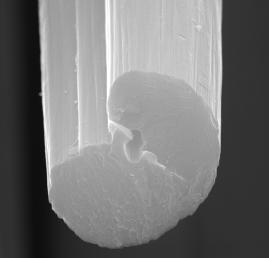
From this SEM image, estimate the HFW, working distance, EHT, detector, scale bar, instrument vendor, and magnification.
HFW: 10.8 µm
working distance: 8.17 mm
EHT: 30 kV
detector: SE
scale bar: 2000 nm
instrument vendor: Tescan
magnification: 26.7 K X